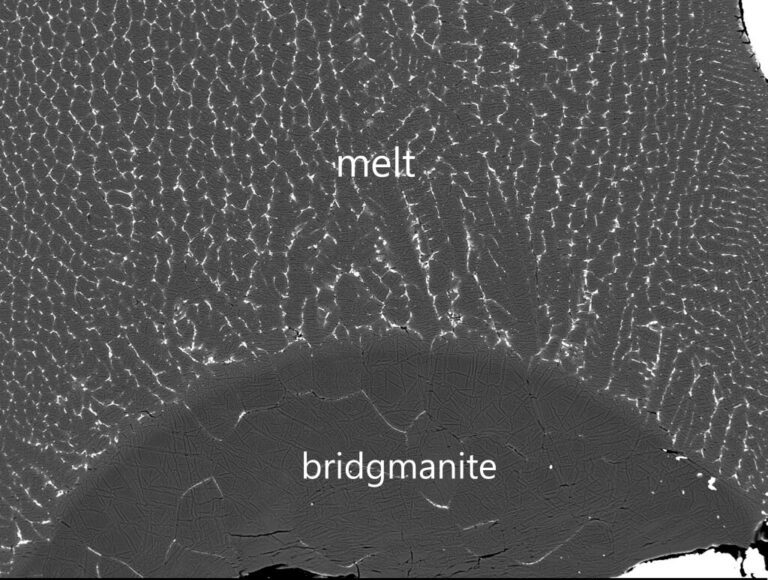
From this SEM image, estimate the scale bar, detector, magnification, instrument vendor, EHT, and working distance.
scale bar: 100000 nm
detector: COMPO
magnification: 0.25 K X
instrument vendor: JEOL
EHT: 15 kV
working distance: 10 mm